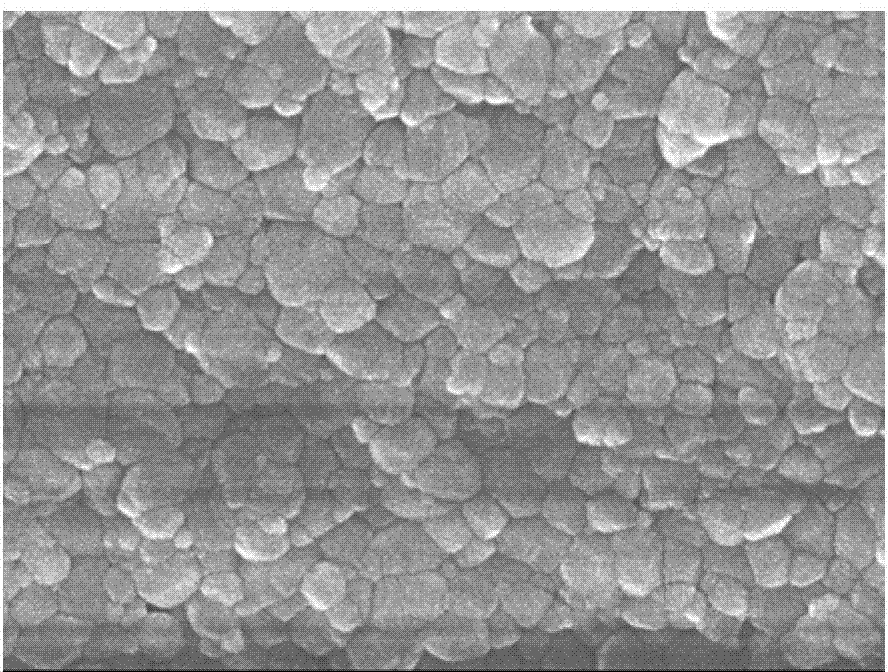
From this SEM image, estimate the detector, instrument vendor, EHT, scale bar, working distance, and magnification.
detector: SEI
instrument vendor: JEOL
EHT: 10 kV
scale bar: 100 nm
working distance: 7.7 mm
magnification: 50 K X